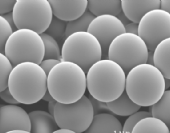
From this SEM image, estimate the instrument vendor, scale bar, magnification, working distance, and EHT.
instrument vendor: JEOL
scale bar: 1000 nm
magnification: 5 K X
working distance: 18 mm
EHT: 5 kV